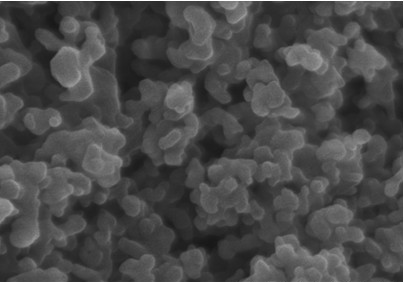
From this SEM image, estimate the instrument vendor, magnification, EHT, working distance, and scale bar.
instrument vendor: Hitachi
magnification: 100 K X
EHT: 15 kV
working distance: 8.2 mm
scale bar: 500 nm